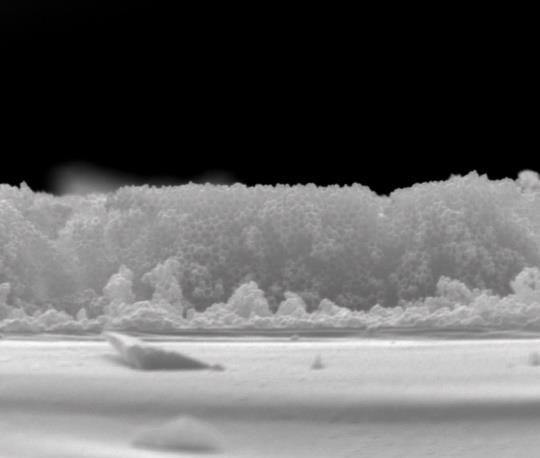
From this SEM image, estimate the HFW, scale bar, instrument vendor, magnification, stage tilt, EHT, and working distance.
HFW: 13.5 µm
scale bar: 5000 nm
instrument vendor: FEI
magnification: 20 K X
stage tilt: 2°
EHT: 25 kV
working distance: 10.4 mm